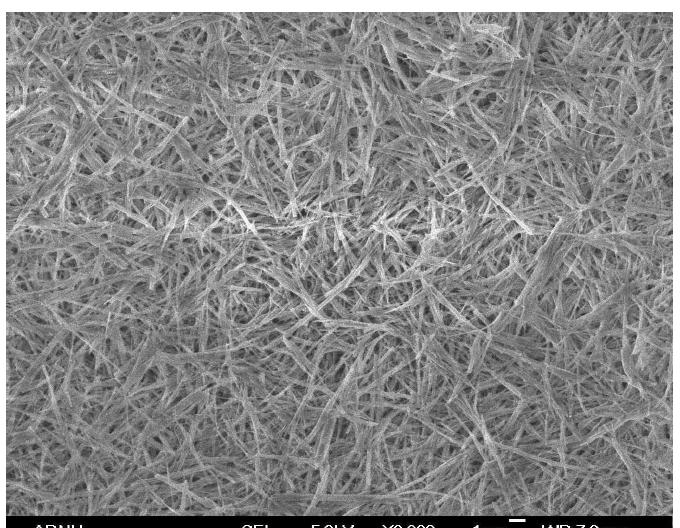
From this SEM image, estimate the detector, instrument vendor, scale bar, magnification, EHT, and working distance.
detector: SEI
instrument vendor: JEOL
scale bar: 1000 nm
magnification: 3 K X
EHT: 5 kV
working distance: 7.8 mm